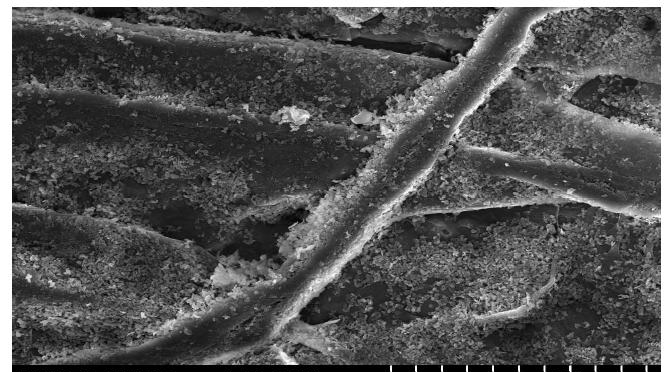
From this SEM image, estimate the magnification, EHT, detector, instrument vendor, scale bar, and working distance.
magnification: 1 K X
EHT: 15 kV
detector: SE(U)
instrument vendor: Hitachi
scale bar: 50000 nm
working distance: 11.9 mm